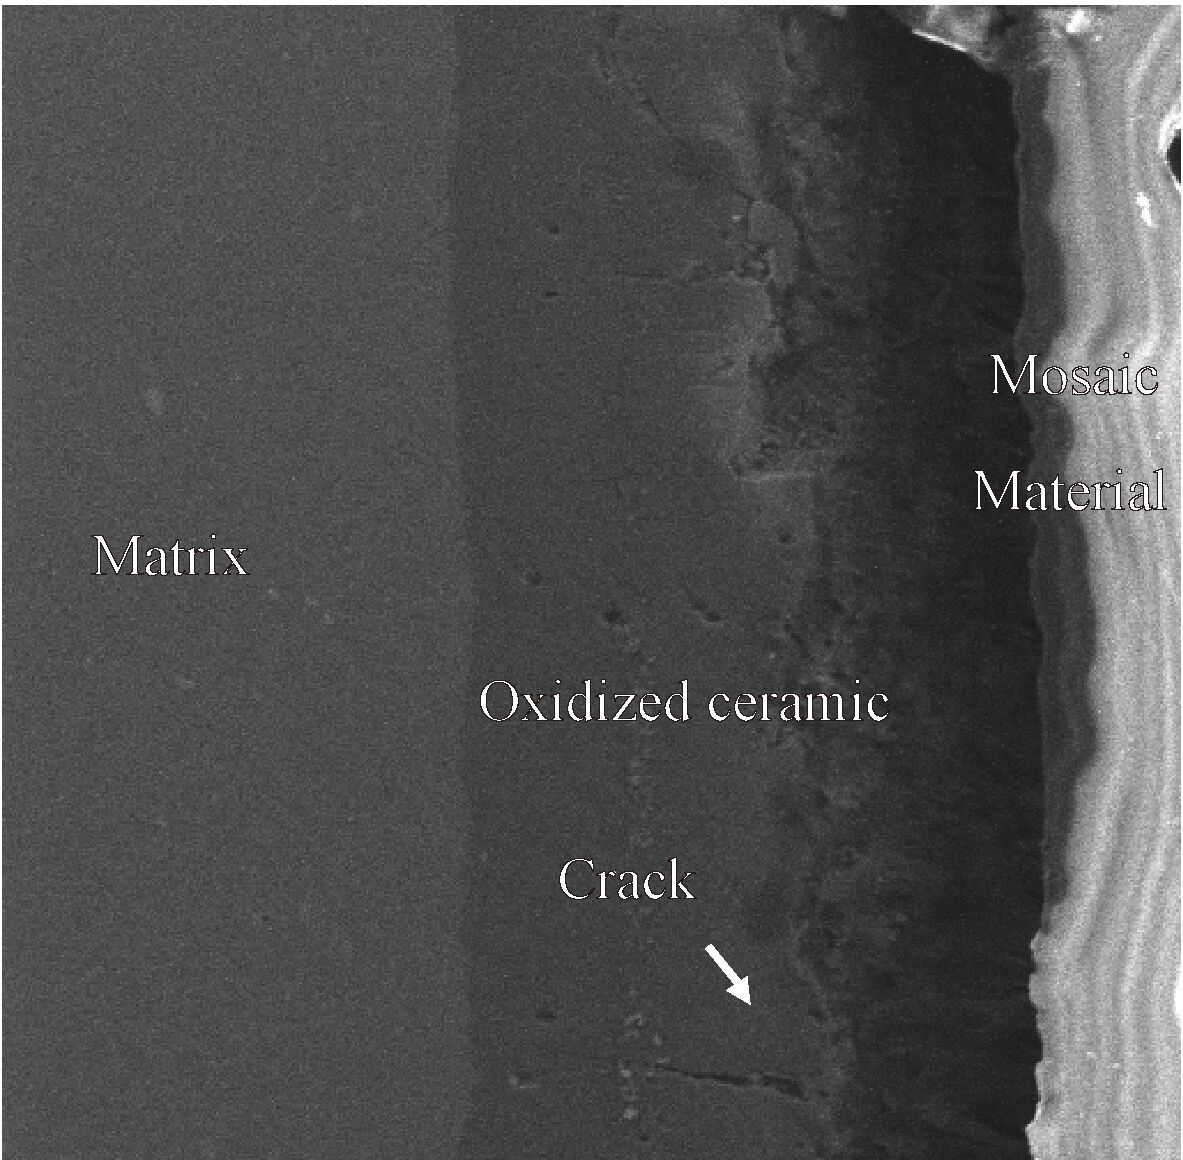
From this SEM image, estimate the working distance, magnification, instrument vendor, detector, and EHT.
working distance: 21.45 mm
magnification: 1 K X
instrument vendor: Tescan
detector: SE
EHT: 10 kV